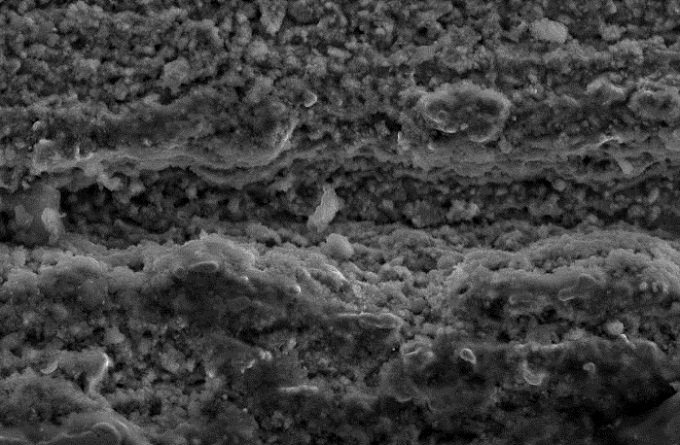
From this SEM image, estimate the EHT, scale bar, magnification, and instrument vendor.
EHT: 20 kV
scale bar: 10000 nm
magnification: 1 K X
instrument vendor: JEOL